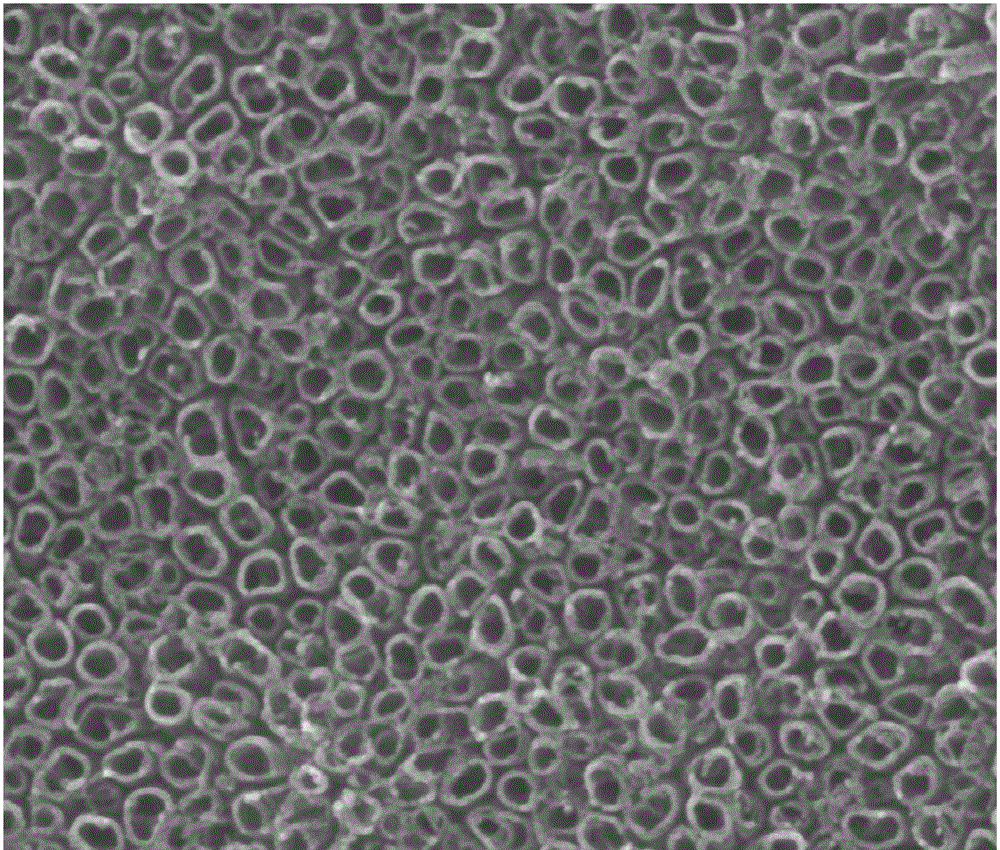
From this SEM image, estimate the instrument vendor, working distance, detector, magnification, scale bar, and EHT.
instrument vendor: FEI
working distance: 9.9 mm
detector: SE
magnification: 100 K X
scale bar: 1000 nm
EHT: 30 kV